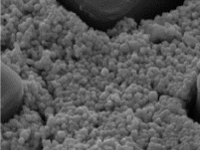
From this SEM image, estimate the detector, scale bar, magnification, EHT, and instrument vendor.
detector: SE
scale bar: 1000 nm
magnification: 50 K X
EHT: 15 kV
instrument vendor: Tescan